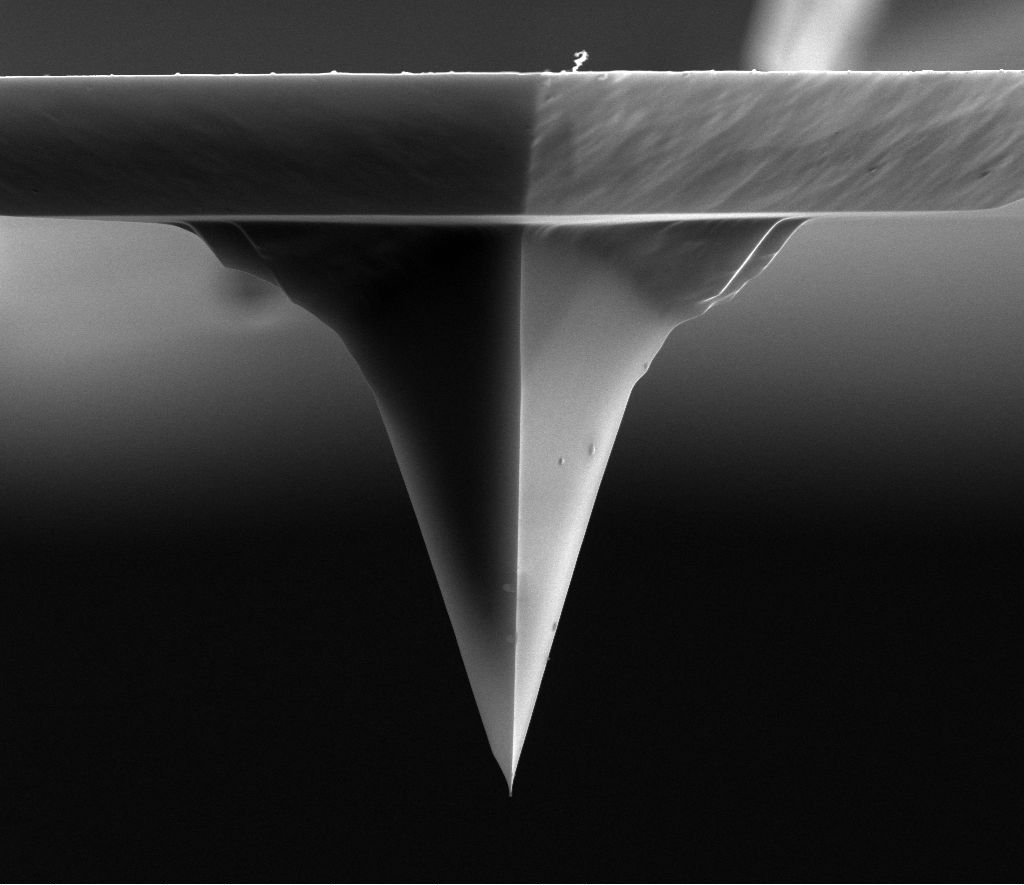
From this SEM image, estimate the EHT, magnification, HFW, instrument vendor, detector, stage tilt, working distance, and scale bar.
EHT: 15 kV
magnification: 5 K X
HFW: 25.6 µm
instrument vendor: FEI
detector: ETD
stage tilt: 0°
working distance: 3.8 mm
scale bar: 10000 nm